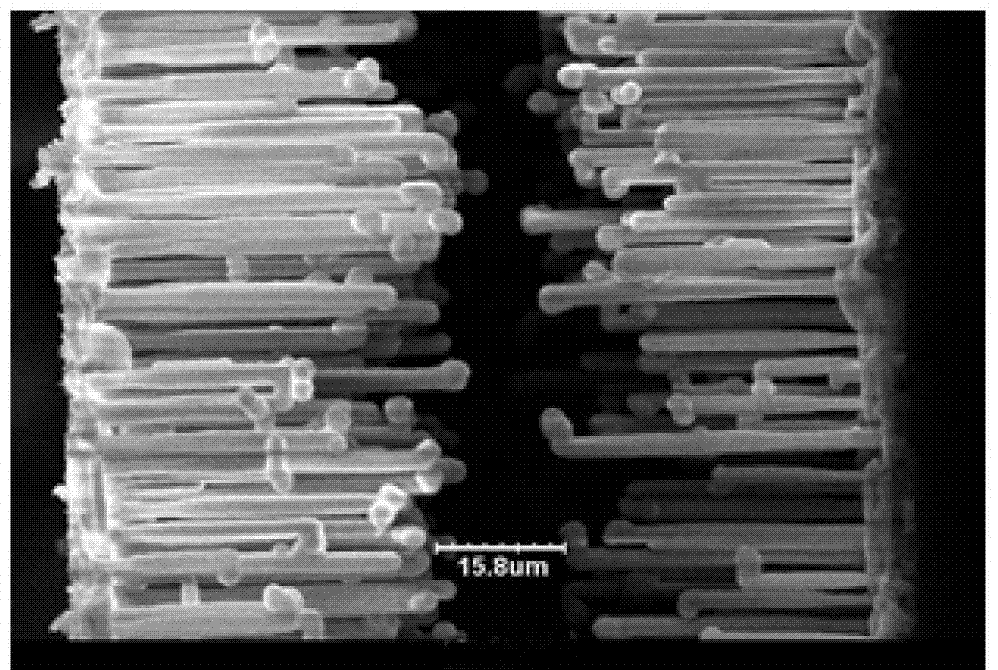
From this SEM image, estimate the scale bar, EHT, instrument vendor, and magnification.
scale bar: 10000 nm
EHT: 15 kV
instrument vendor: other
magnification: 1 K X